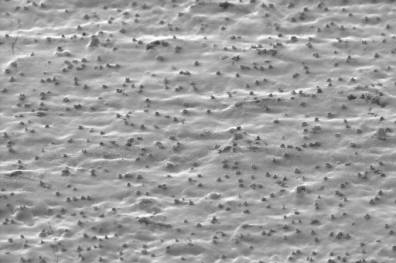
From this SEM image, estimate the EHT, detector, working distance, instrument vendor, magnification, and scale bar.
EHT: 10 kV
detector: SE2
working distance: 10 mm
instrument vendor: Zeiss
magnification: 0.148 K X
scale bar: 100000 nm